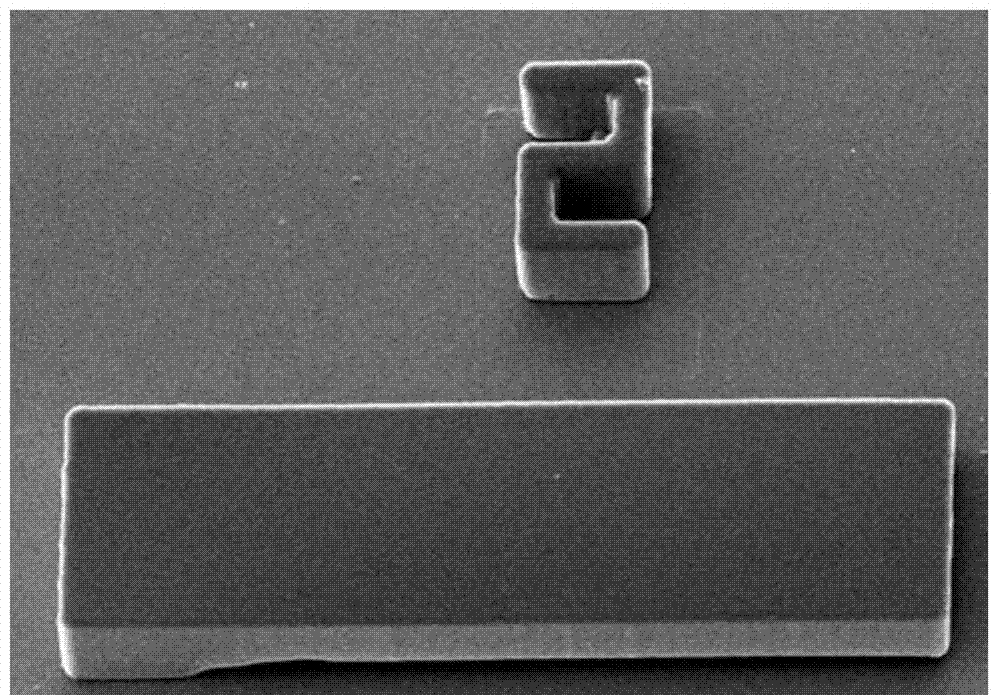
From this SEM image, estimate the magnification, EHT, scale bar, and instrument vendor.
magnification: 0.33 K X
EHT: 15 kV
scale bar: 50000 nm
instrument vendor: JEOL